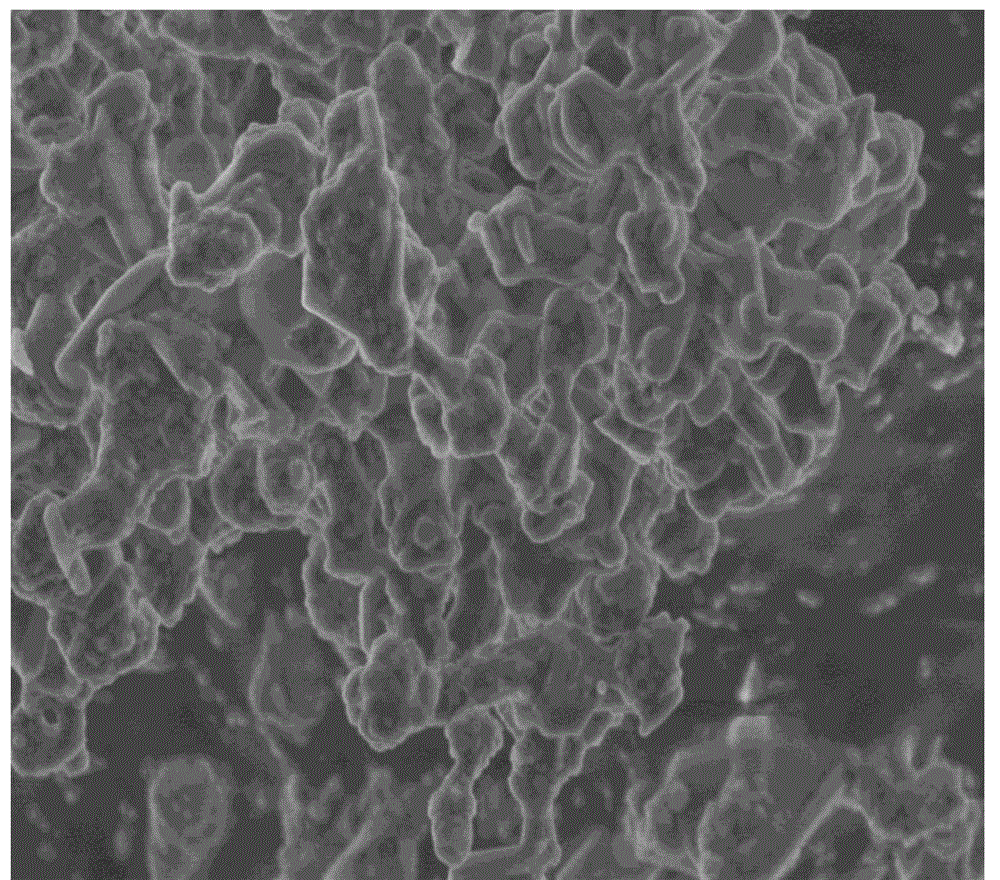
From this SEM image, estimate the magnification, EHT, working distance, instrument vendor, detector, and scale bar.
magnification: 15 K X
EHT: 3 kV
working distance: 7.6 mm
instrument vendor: Hitachi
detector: SE(U)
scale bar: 3000 nm